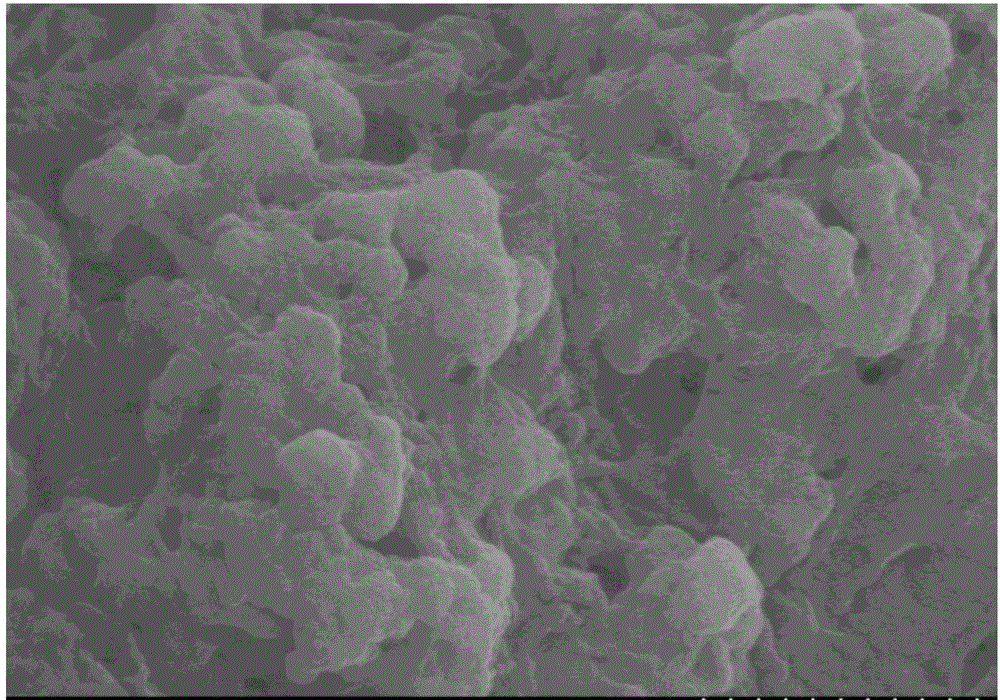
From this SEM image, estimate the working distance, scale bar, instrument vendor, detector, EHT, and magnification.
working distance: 12.7 mm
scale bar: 1000 nm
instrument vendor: Hitachi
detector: SE(M)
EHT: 5 kV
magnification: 35 K X